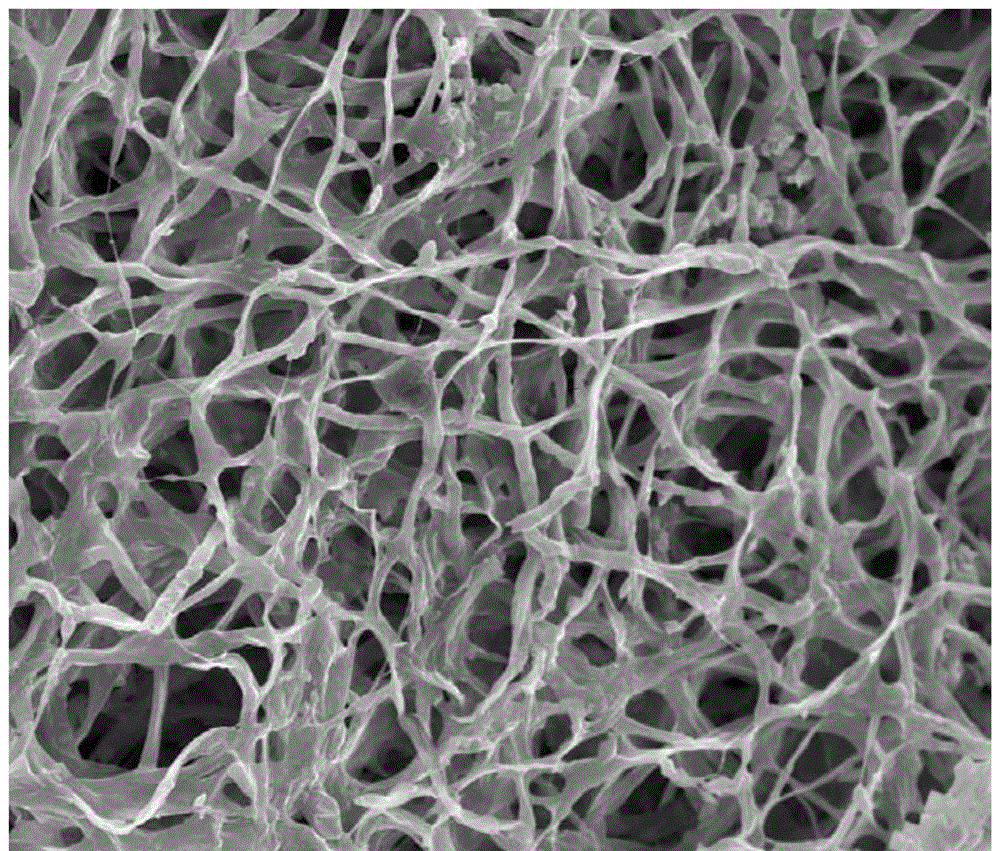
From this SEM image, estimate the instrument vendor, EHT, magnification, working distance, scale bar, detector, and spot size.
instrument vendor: FEI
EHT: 20 kV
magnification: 2 K X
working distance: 10.6 mm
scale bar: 50000 nm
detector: ETD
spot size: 4.5